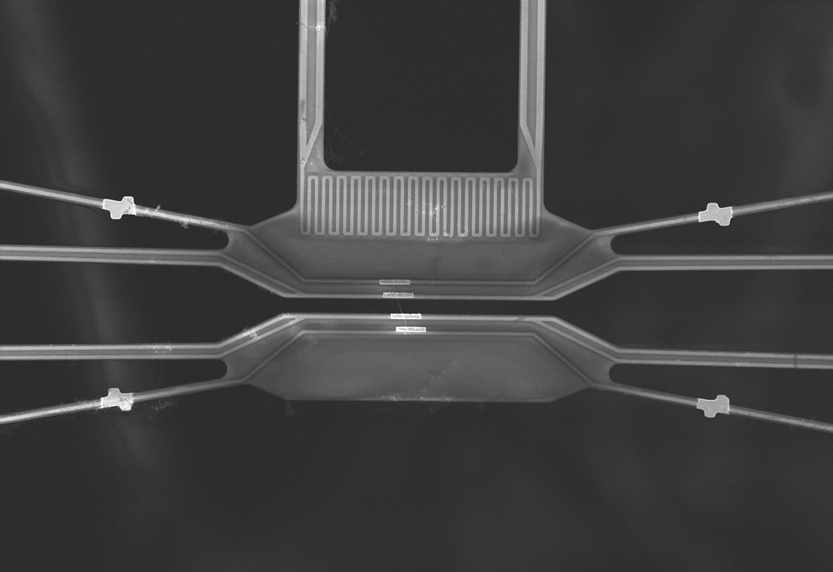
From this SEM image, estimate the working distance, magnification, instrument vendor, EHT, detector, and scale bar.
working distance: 8.8 mm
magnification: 0.9 K X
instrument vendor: Hitachi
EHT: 10 kV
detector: SE(U)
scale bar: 50000 nm